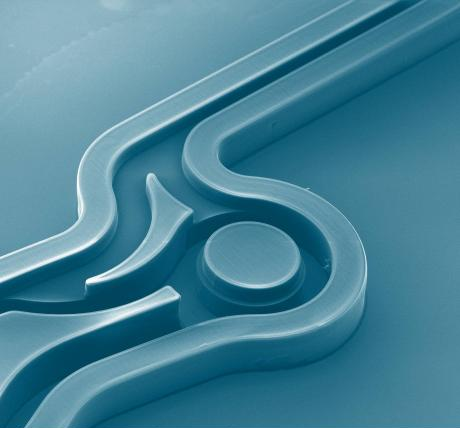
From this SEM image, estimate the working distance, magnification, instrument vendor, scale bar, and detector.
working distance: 16.4 mm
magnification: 0.083 K X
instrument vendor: FEI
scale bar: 1000000 nm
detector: ETD